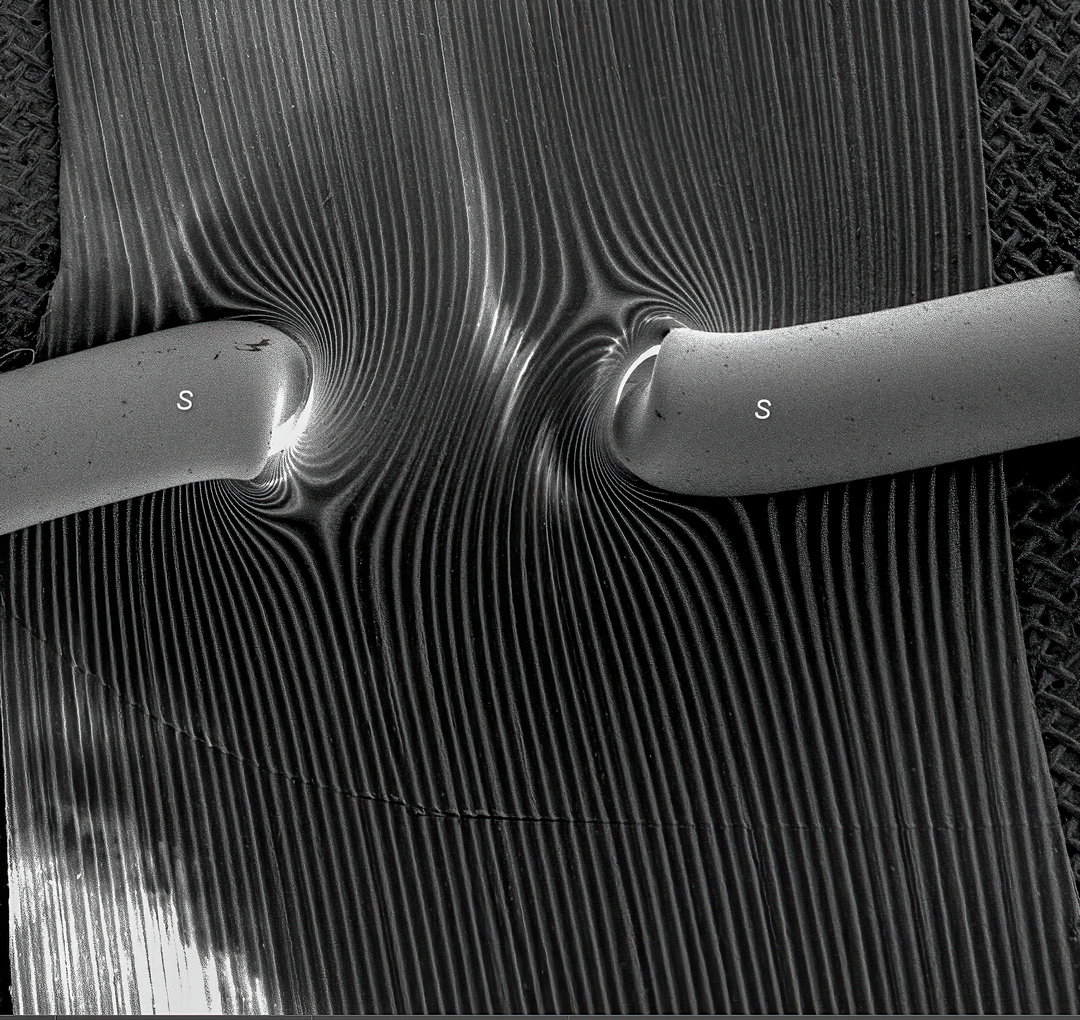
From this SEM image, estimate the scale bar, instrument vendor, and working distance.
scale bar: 5000000 nm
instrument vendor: FEI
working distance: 69.73 mm